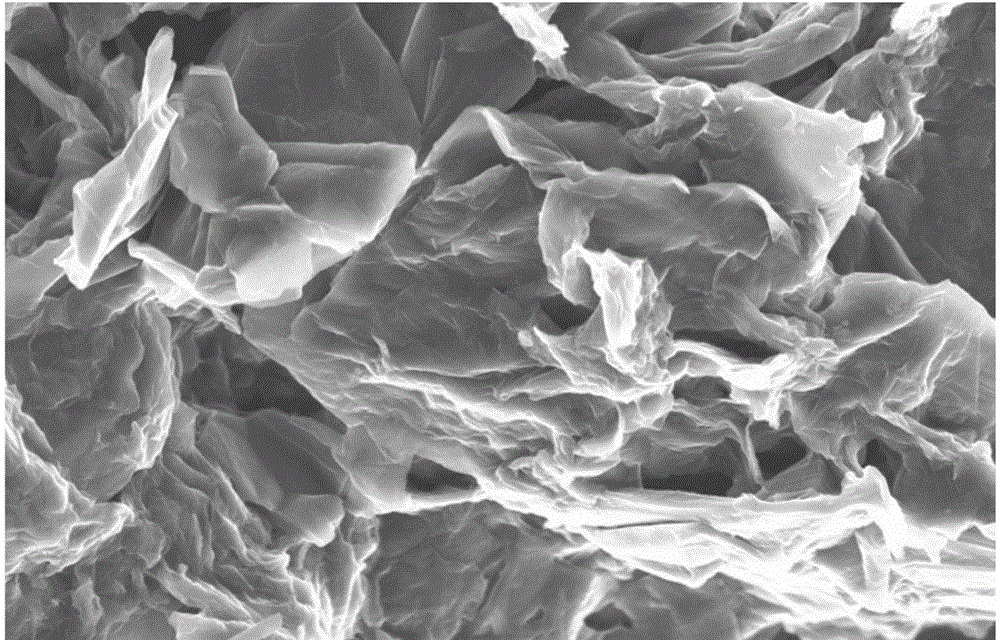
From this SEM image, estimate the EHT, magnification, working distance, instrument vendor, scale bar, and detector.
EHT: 5 kV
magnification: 7 K X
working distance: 5 mm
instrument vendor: JEOL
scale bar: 1000 nm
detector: SEI